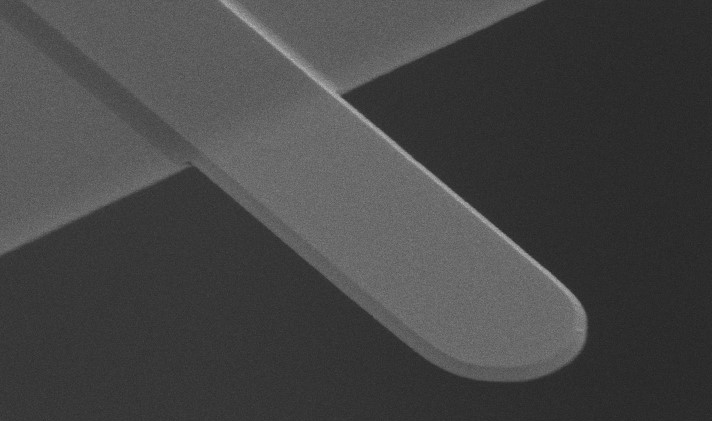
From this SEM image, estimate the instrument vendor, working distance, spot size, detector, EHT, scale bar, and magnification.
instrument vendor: FEI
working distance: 8.6 mm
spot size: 3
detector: SE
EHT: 10 kV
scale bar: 2000 nm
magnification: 7.993 K X